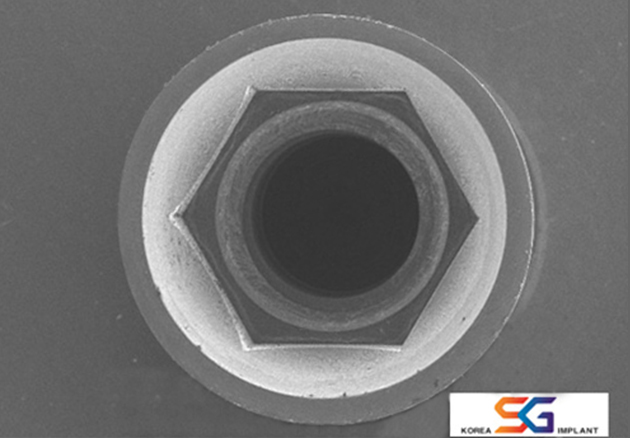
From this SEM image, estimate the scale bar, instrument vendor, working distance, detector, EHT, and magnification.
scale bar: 2000000 nm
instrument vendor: Hitachi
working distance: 42.3 mm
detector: SE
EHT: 15 kV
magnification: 0.02 K X